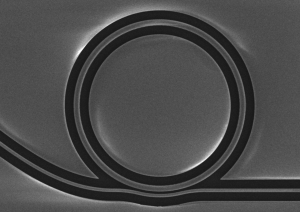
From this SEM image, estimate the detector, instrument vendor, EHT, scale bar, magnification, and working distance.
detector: LED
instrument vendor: JEOL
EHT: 15 kV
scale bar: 10000 nm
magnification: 1.5 K X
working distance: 6.8 mm